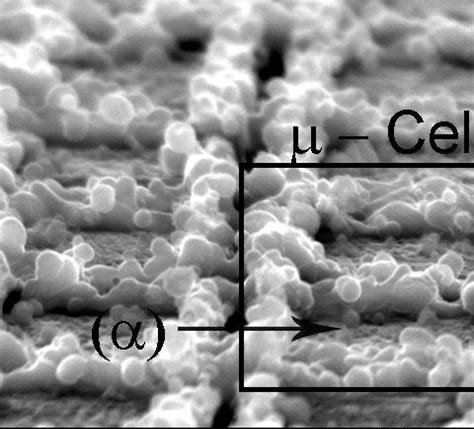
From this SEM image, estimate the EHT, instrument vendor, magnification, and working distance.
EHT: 25 kV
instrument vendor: JEOL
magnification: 2.5 K X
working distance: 22.7 mm